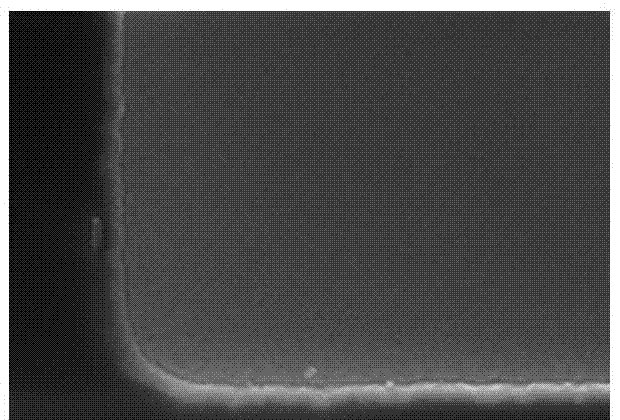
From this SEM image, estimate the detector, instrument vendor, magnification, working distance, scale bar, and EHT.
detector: SE(U)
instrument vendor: Hitachi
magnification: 100 K X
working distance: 9.6 mm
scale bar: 500 nm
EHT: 15 kV